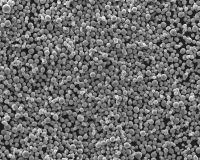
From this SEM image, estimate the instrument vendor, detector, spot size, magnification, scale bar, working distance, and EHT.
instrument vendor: FEI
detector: ETD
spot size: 3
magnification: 1 K X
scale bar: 100000 nm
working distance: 11.2 mm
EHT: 25 kV